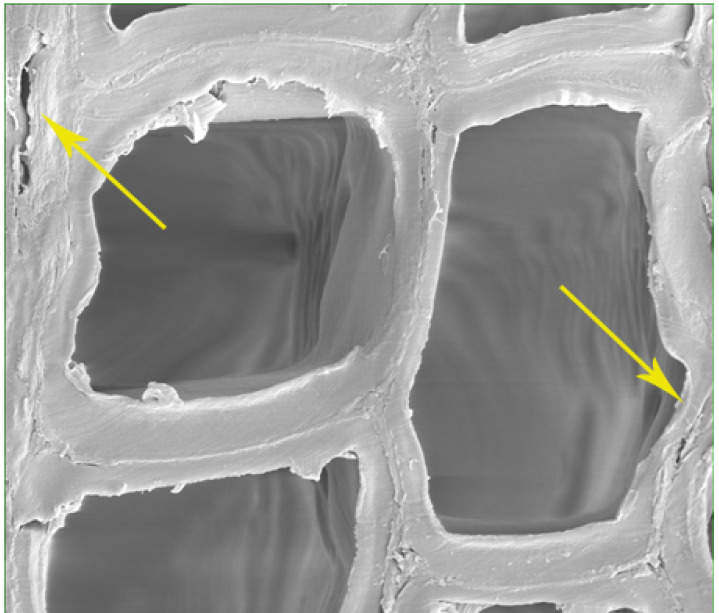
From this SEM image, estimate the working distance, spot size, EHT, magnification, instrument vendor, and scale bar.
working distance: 10.7 mm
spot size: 4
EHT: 7 kV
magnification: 5 K X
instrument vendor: FEI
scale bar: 30000 nm